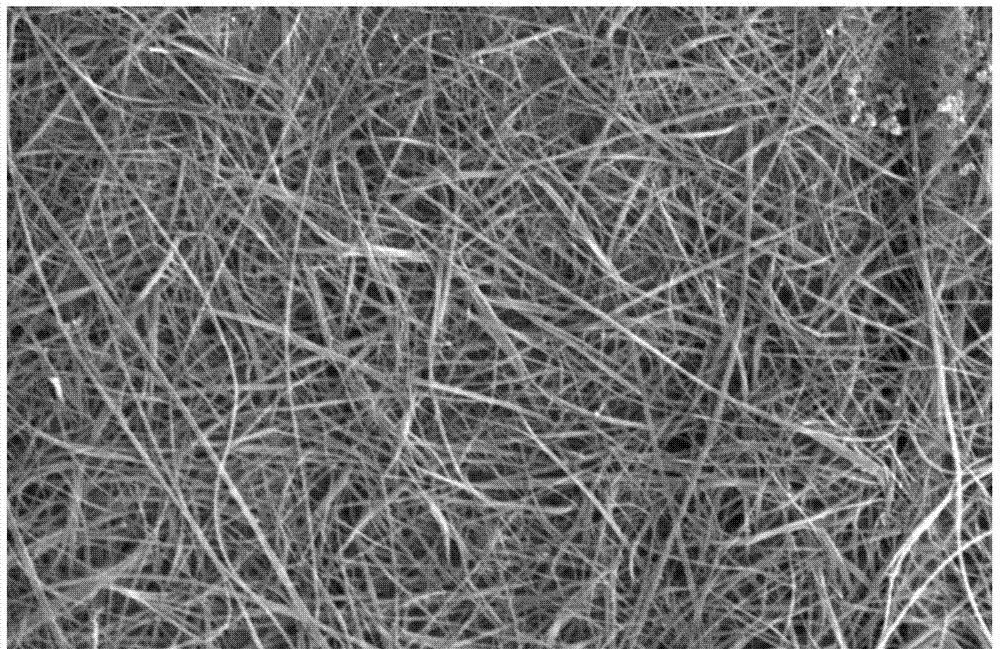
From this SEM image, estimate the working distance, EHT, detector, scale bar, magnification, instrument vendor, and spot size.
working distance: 4.8 mm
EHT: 25 kV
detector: SE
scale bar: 5000 nm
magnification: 5 K X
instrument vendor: FEI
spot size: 4